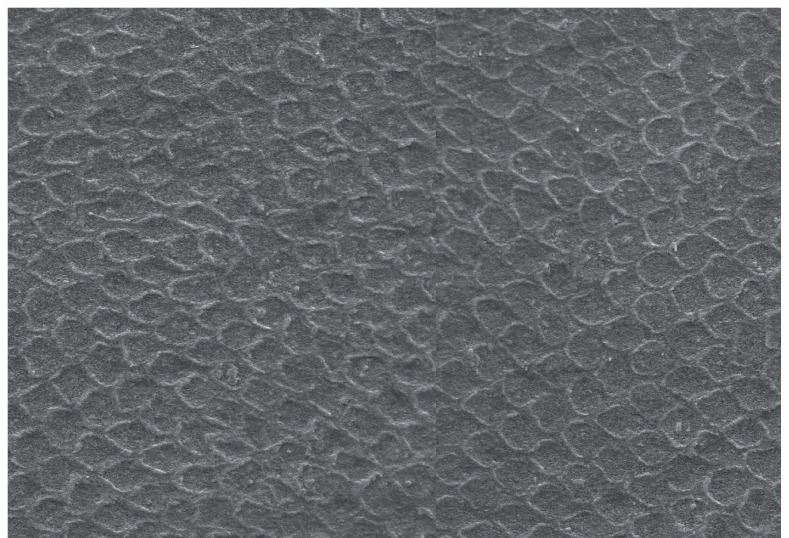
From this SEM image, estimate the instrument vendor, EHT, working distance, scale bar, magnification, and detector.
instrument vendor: Zeiss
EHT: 20 kV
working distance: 12 mm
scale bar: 10000 nm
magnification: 2.5 K X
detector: SE1